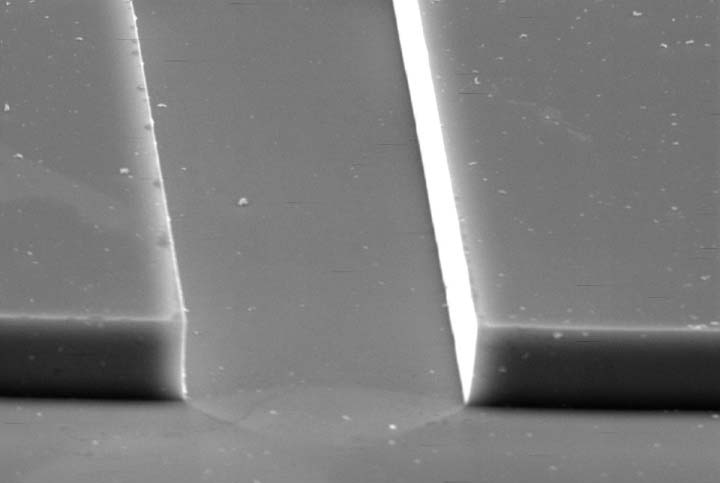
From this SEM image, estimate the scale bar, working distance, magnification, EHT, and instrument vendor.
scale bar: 20000 nm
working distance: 48 mm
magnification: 1 K X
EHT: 25 kV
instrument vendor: Zeiss